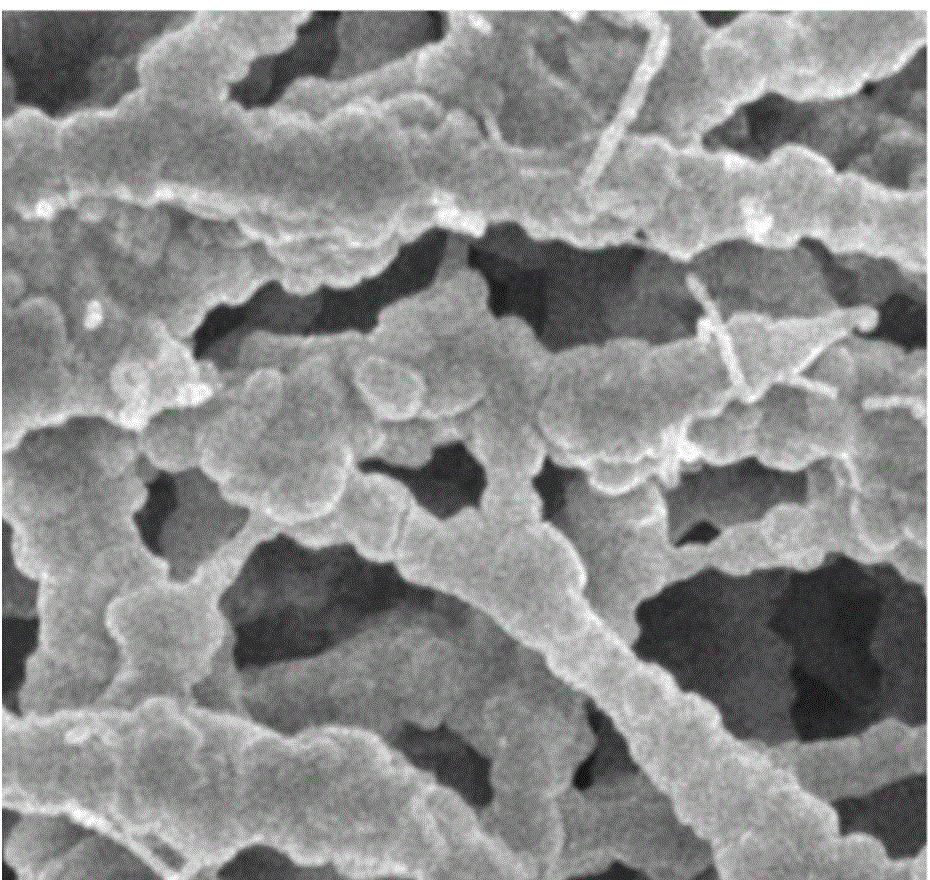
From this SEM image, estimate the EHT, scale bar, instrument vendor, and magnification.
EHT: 5 kV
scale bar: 1000 nm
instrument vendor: JEOL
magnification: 10 K X